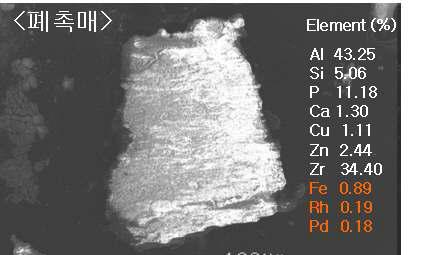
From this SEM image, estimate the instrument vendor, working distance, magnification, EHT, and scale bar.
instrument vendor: JEOL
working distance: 14 mm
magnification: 0.08 K X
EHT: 20 kV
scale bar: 100000 nm